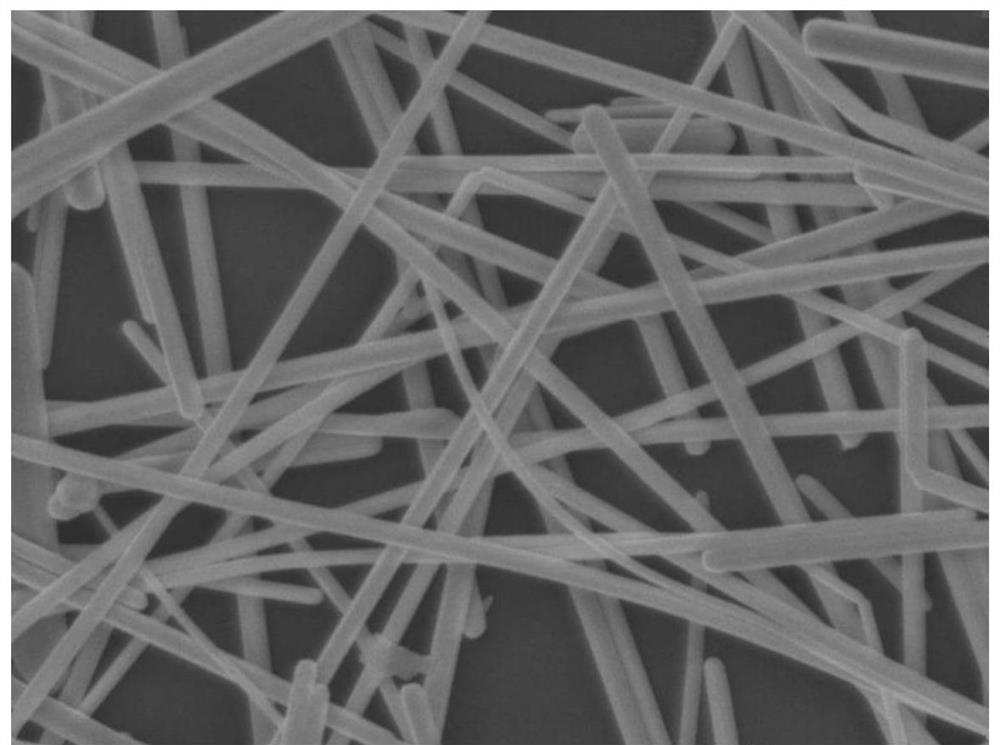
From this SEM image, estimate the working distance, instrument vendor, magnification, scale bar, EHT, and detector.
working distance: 6 mm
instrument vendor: JEOL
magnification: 30 K X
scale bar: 100 nm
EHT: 5 kV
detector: SEI